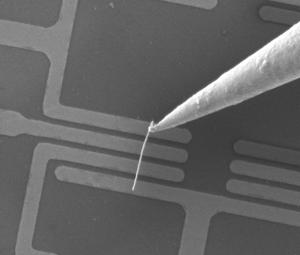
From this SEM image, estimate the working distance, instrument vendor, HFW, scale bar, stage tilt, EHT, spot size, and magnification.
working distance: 4.99 mm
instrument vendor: FEI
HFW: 60.7 µm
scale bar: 10000 nm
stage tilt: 0°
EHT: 10 kV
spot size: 2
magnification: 5.01 K X